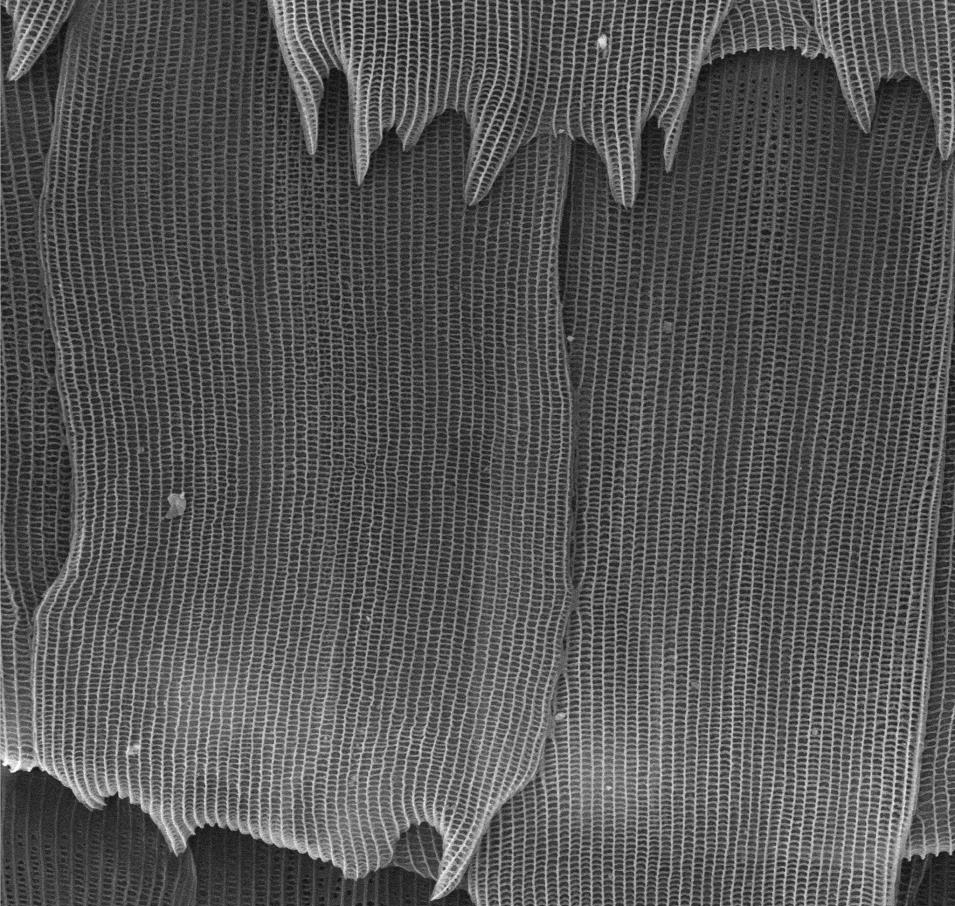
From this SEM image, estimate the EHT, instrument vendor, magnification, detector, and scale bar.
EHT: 15 kV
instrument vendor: other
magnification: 3 K X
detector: SE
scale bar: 10000 nm